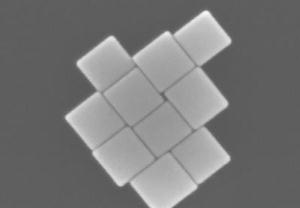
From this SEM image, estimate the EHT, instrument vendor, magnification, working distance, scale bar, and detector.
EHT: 10 kV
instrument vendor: Hitachi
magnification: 350 K X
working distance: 5.9 mm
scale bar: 100 nm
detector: SE(U)